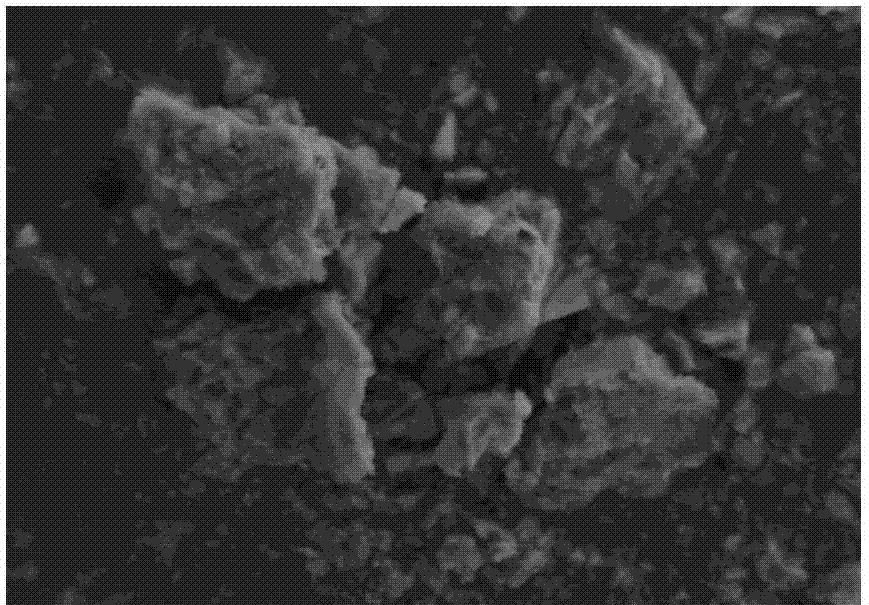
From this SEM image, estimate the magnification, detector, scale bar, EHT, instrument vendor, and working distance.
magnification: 10 K X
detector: SE2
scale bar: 1000 nm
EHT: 5 kV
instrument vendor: Zeiss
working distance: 5.6 mm